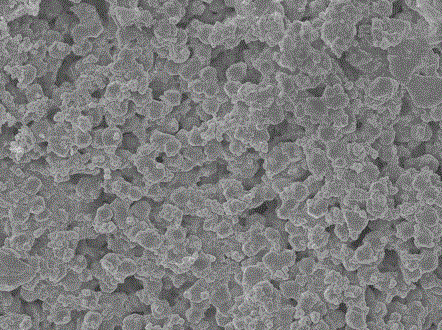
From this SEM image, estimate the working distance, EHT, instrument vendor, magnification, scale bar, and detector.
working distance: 7.8 mm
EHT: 5 kV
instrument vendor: JEOL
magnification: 3 K X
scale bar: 1000 nm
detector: SEI